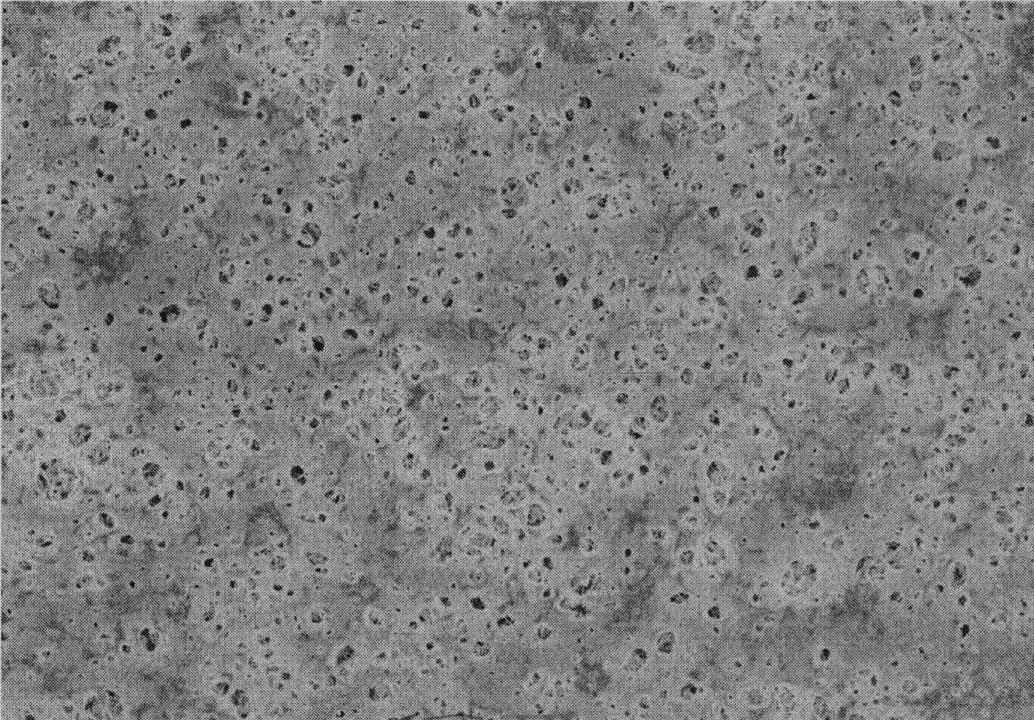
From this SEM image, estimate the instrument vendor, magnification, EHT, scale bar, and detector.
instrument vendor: Hitachi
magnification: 1 K X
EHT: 4 kV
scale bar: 50000 nm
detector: SE(M)